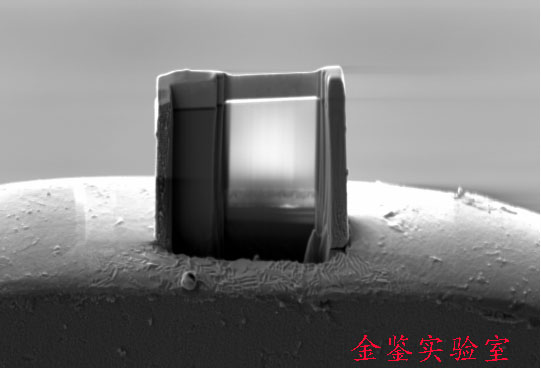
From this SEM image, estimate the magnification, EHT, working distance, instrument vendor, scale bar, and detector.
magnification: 3.92 K X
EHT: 5 kV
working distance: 5.2 mm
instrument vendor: Zeiss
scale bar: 1000 nm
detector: SE2